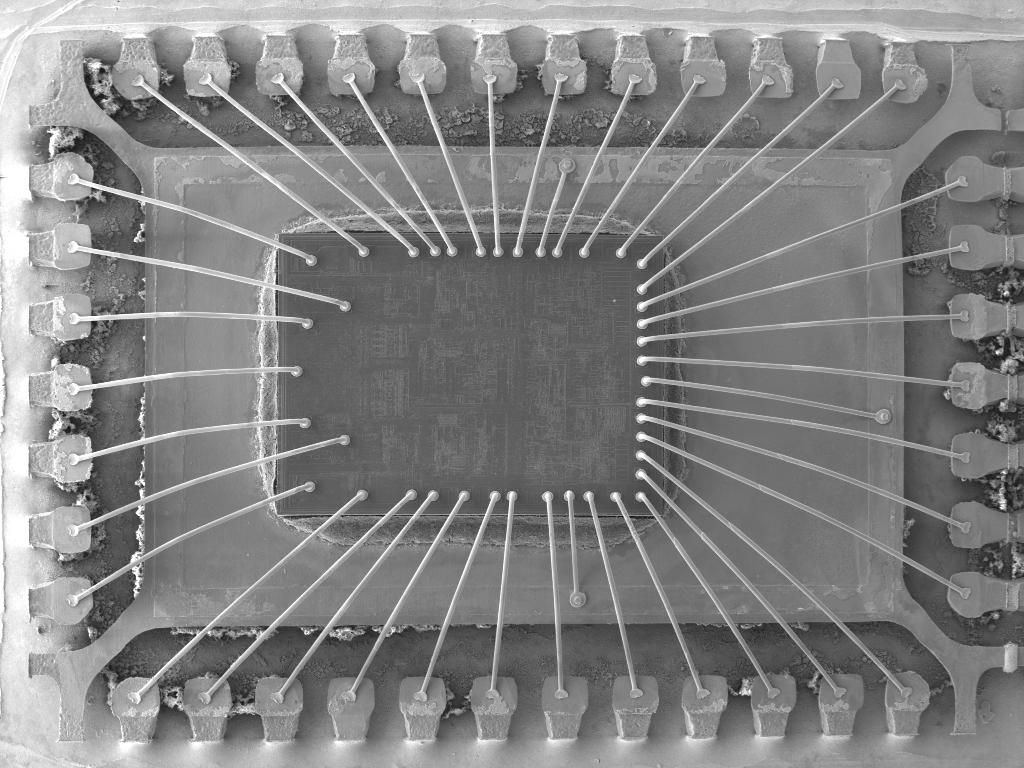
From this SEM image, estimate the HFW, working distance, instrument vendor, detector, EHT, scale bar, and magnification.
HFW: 6880 µm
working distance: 15.01 mm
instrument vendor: Tescan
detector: SE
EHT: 20 kV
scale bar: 2000000 nm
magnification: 0.04 K X